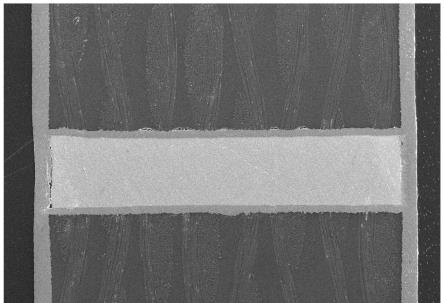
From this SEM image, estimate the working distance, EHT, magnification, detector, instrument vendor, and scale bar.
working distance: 6.8 mm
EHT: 20 kV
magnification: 0.07 K X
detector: SE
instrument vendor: Hitachi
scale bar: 500000 nm